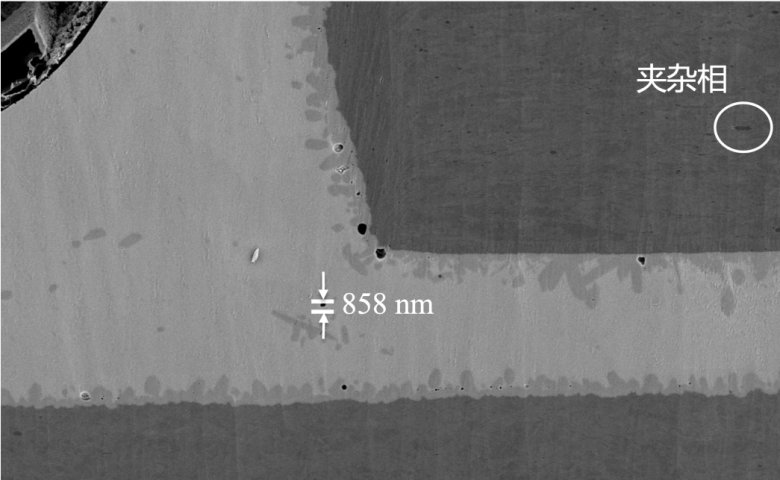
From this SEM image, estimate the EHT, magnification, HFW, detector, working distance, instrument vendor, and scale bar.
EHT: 10 kV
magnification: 1 K X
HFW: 127 µm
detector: Mix 50%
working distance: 7.491 mm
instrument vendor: Thermo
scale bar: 30000 nm